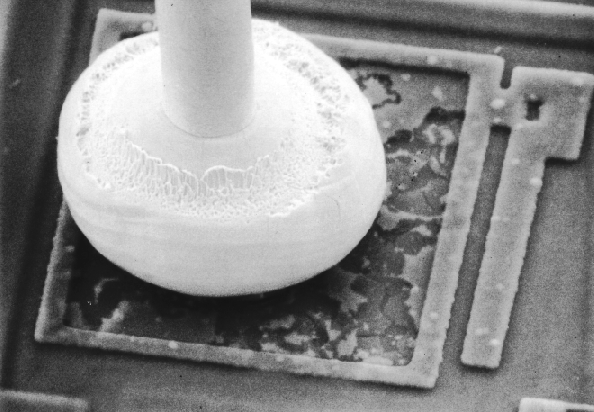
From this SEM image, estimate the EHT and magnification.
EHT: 30 kV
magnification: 0.56 K X